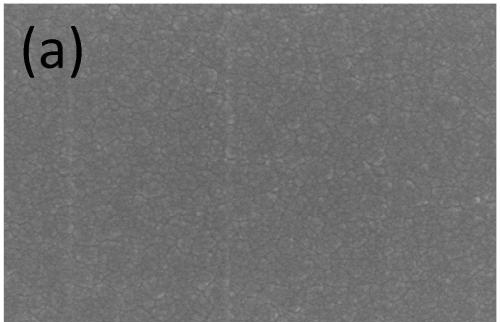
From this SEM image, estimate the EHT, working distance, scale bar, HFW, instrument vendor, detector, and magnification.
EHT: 2 kV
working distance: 4 mm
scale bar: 500 nm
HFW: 4.23 µm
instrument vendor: Thermo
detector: TLD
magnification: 30 K X